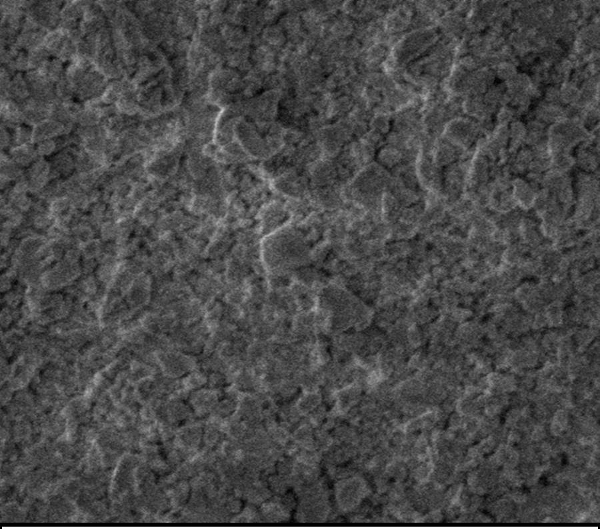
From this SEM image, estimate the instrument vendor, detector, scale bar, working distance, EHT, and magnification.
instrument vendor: Zeiss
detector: InLens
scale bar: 300 nm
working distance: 8.8 mm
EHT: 2 kV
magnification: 50.53 K X